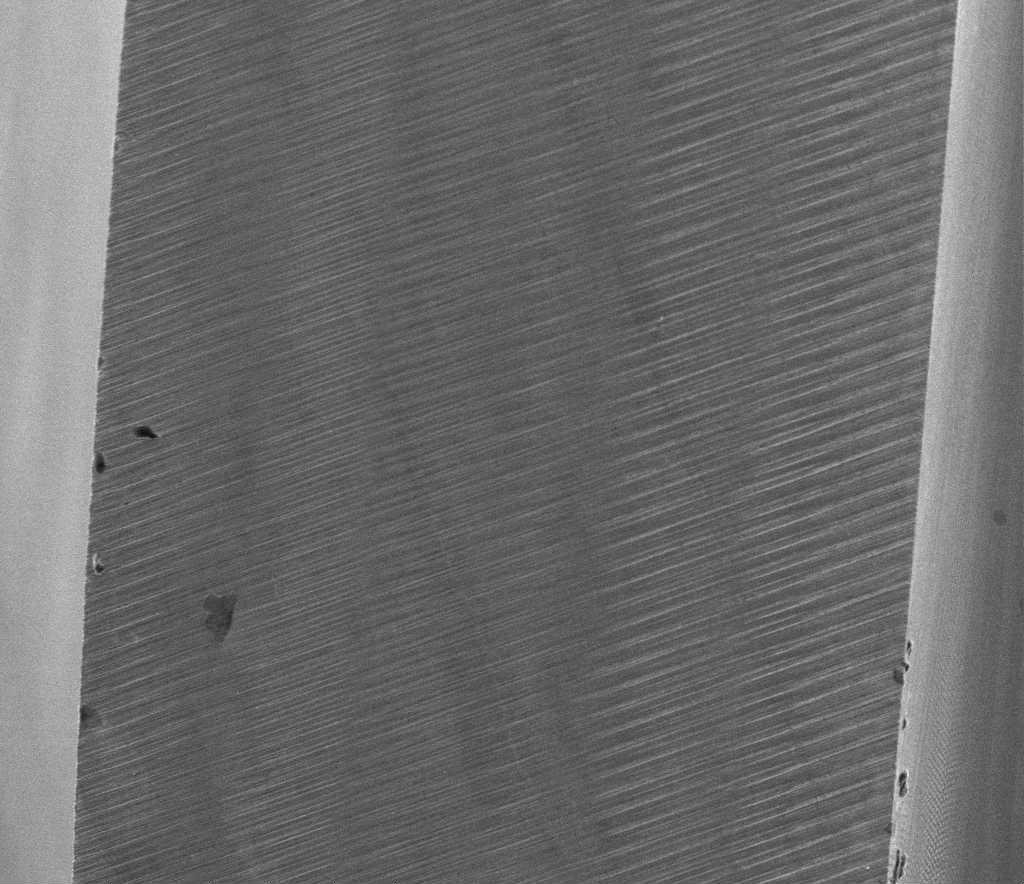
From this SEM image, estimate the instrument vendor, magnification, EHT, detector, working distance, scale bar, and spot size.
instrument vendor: FEI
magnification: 0.08 K X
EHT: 20 kV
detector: ETD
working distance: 12.1 mm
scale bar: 500000 nm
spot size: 3.5